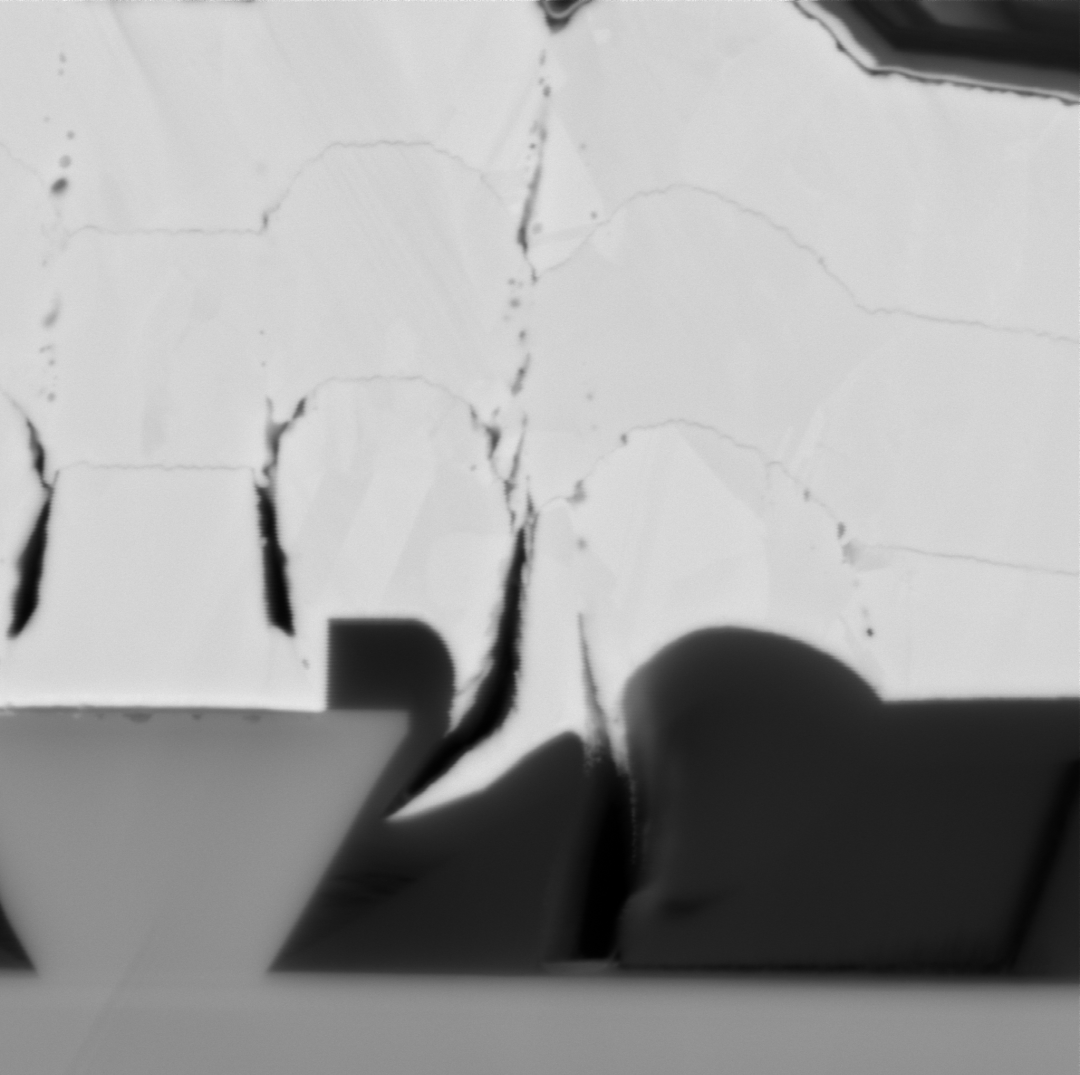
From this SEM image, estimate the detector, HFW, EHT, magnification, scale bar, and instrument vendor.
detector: BSD Full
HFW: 6.71 µm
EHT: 15 kV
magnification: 40 K X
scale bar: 2000 nm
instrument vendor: other